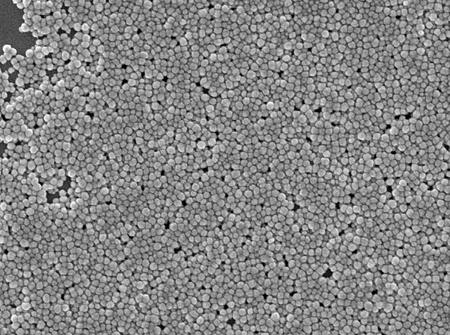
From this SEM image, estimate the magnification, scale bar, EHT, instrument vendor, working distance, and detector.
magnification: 45 K X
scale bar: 100 nm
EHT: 3 kV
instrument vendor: JEOL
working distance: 4.3 mm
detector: SEI